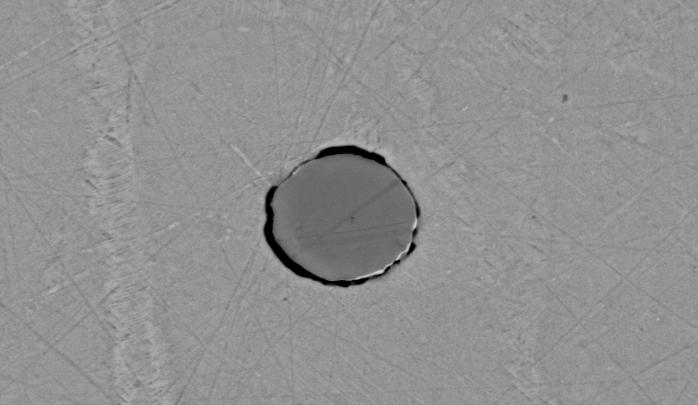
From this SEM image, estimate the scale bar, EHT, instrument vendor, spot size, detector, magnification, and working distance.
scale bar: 10000 nm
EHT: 20 kV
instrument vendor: FEI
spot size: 4.5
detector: BSE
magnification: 2 K X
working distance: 11.3 mm